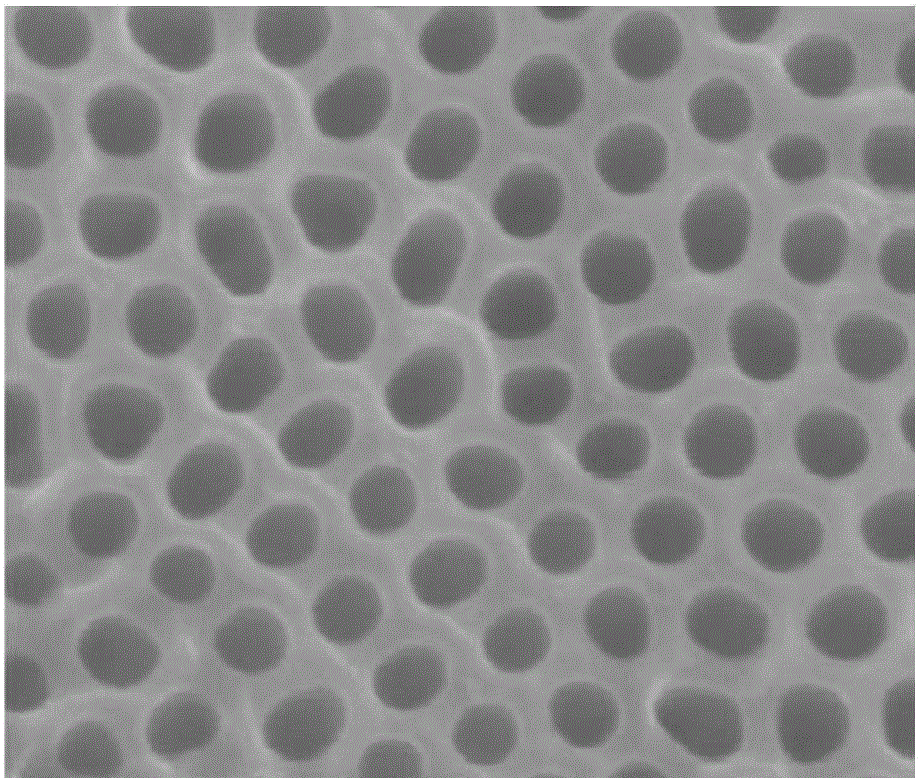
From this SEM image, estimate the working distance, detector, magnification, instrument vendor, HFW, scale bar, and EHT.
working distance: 4.9 mm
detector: TLD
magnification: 200 K X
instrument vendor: FEI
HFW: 1.2 µm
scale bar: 300 nm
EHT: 10 kV